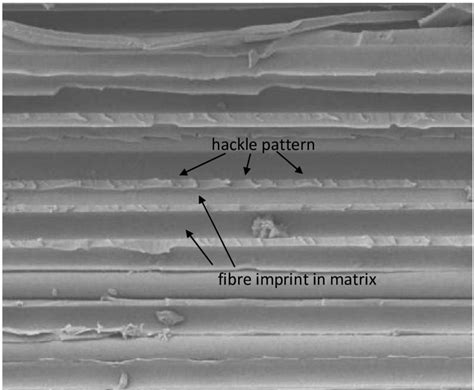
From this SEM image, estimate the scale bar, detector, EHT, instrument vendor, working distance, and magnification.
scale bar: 10000 nm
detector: LEI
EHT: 5 kV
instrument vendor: JEOL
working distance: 14 mm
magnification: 1 K X